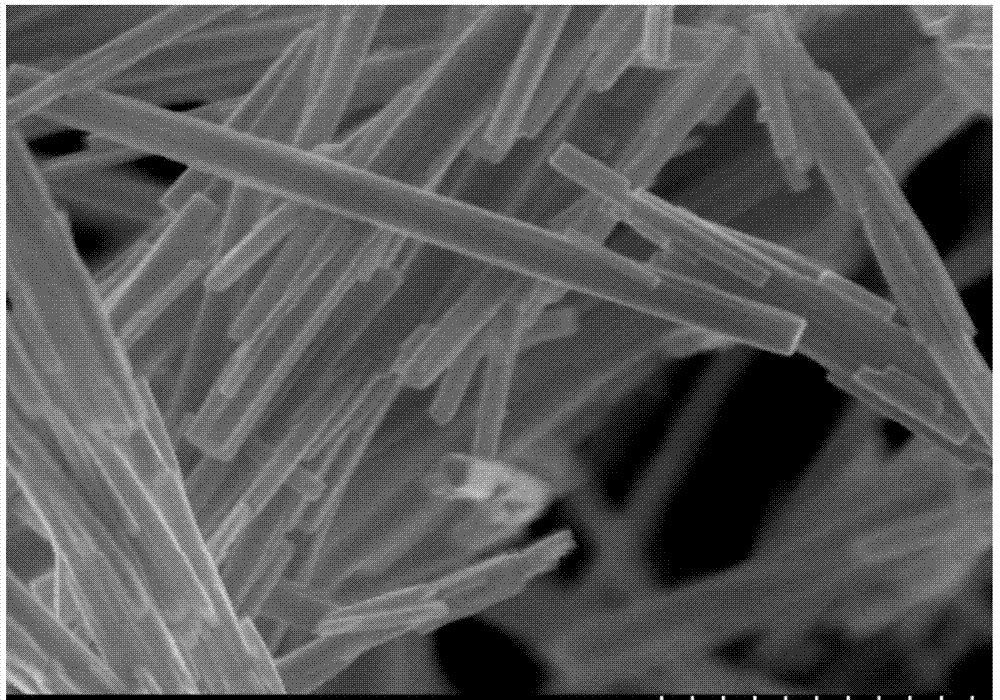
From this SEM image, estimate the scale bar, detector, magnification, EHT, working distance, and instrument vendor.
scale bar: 500 nm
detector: SE(U)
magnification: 80 K X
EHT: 5 kV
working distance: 3.4 mm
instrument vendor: Hitachi